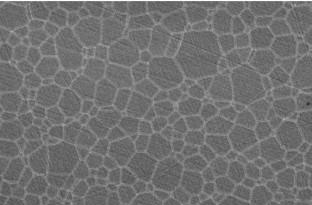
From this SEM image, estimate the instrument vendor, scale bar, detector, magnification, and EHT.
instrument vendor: JEOL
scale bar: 20000 nm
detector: SEI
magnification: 0.8 K X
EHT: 15 kV